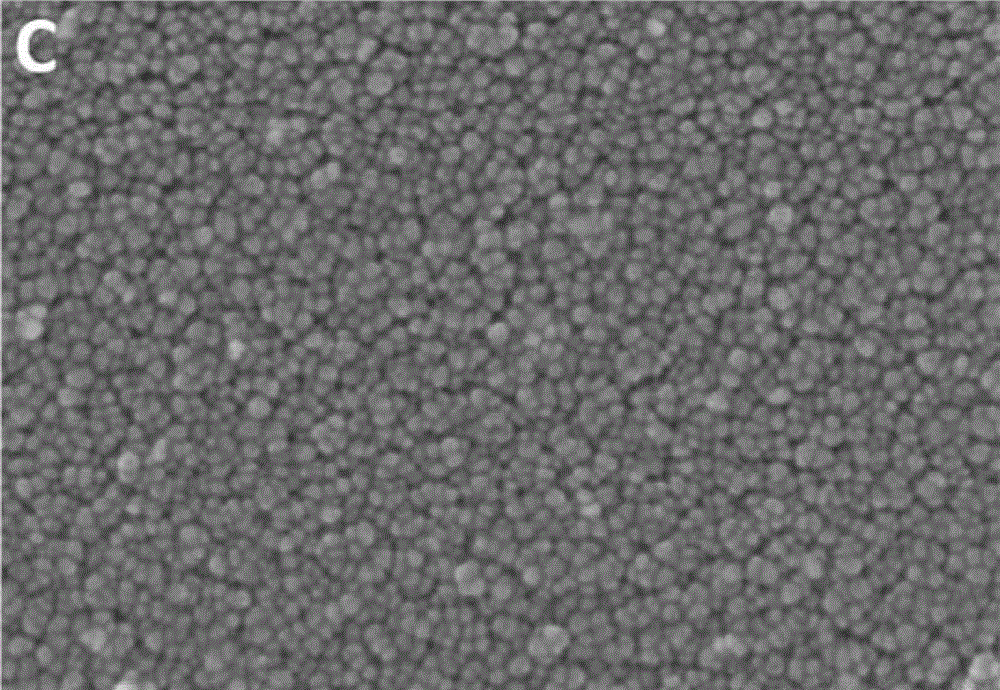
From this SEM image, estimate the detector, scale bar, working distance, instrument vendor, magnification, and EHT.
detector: SE(U)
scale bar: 100 nm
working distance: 8 mm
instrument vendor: Hitachi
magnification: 300 K X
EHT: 15 kV